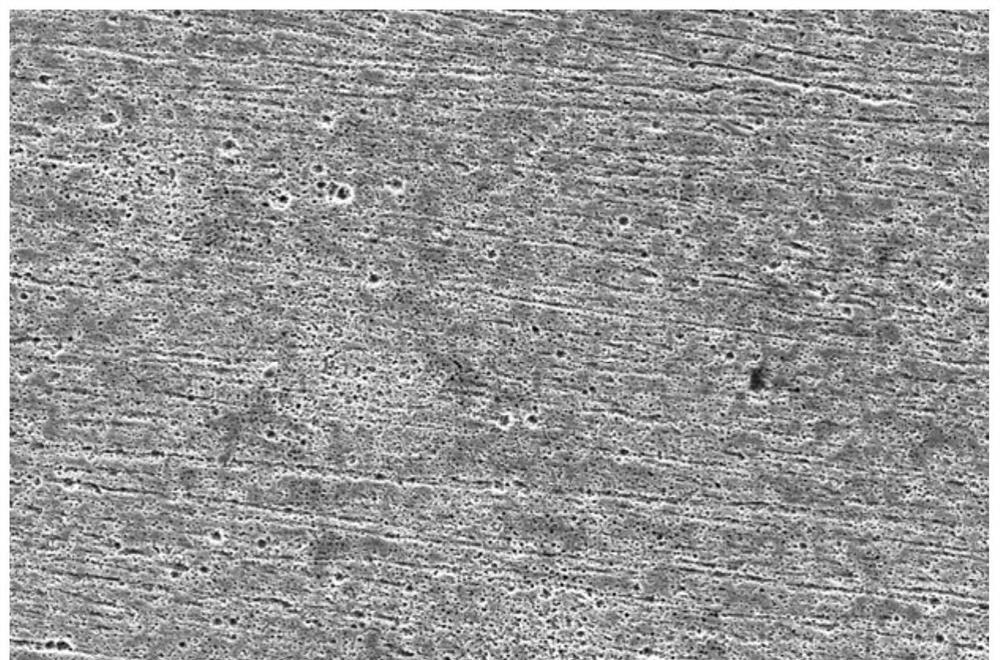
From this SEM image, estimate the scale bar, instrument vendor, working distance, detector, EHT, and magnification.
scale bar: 50000 nm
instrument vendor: FEI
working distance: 15.1 mm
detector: SE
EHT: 20 kV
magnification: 1 K X